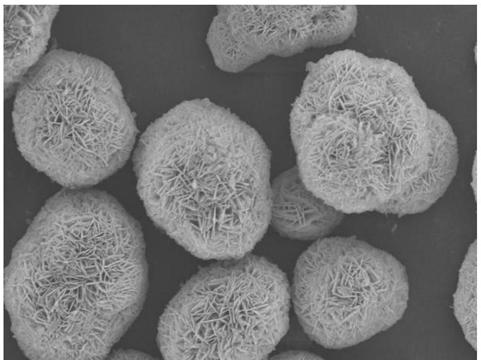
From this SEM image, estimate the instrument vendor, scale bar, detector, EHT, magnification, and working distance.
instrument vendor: JEOL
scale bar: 1000 nm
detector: SEI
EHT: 10 kV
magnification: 5 K X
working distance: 7.6 mm